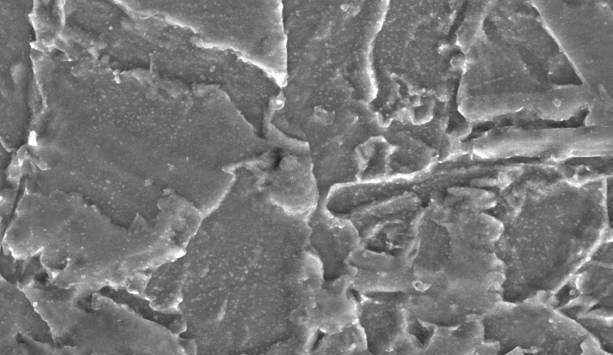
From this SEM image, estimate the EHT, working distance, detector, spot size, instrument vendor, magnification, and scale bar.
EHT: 20 kV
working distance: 11.6 mm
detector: SE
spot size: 4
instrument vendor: FEI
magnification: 3 K X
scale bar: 5000 nm